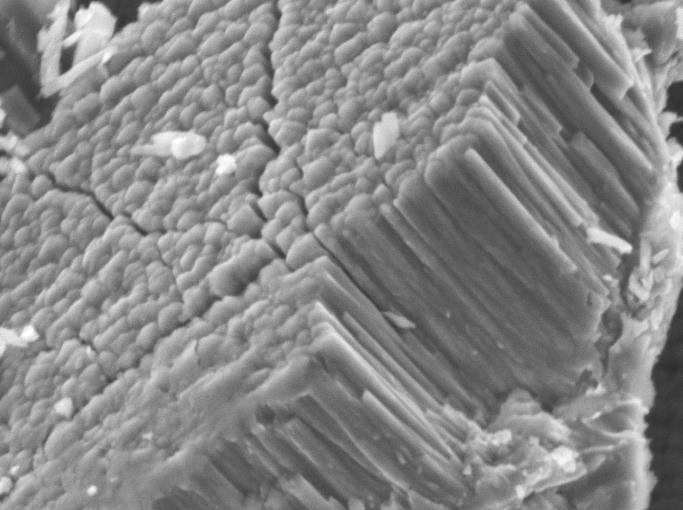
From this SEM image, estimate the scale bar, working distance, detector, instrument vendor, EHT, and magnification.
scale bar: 1000 nm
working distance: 11 mm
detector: SEI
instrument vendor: JEOL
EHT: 7 kV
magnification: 15 K X